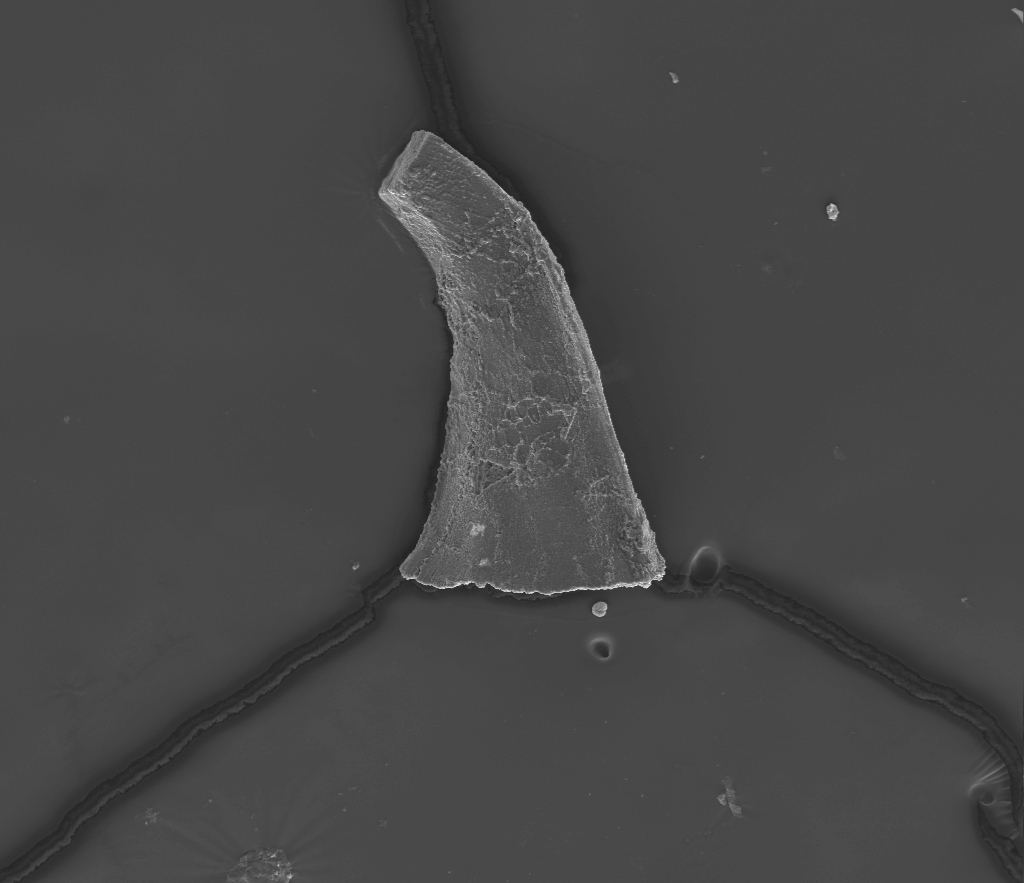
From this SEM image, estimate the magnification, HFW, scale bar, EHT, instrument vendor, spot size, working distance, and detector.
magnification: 0.286 K X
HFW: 950 µm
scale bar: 200000 nm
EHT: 20 kV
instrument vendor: FEI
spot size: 5.5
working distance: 10.2 mm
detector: ETD